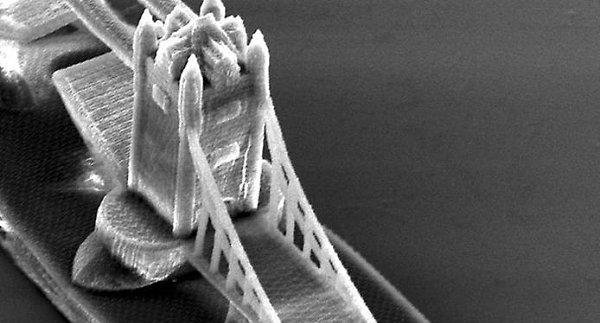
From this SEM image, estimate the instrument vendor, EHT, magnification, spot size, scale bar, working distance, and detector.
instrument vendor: FEI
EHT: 15 kV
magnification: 0.969 K X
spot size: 4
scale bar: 20000 nm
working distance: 27.1 mm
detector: SE